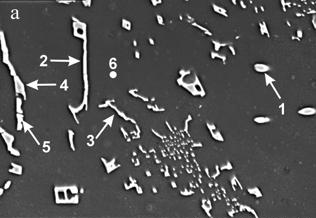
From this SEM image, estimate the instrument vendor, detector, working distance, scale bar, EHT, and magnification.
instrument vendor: Hitachi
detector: SE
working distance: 15.2 mm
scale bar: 30000 nm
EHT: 15 kV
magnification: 1.5 K X